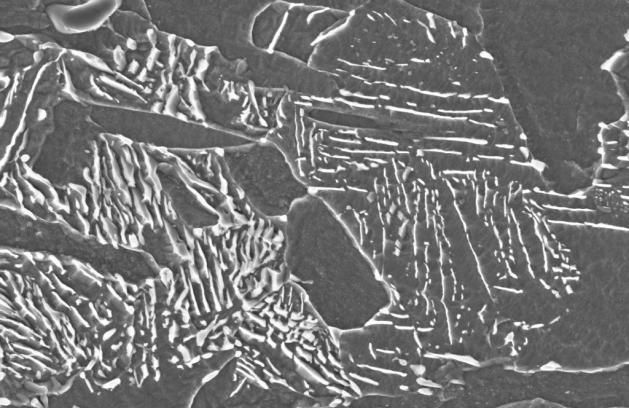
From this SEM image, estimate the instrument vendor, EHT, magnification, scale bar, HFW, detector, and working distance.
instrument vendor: Zeiss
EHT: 5 kV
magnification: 30 K X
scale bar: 1000 nm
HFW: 9.932 µm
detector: InLens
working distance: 2 mm